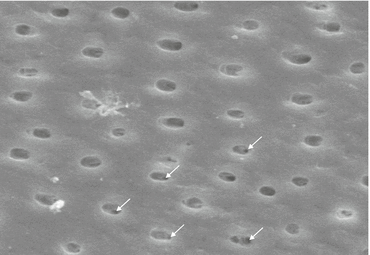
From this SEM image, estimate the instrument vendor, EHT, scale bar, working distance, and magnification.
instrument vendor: Zeiss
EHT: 20 kV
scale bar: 10000 nm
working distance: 22.6 mm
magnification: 3.996 K X